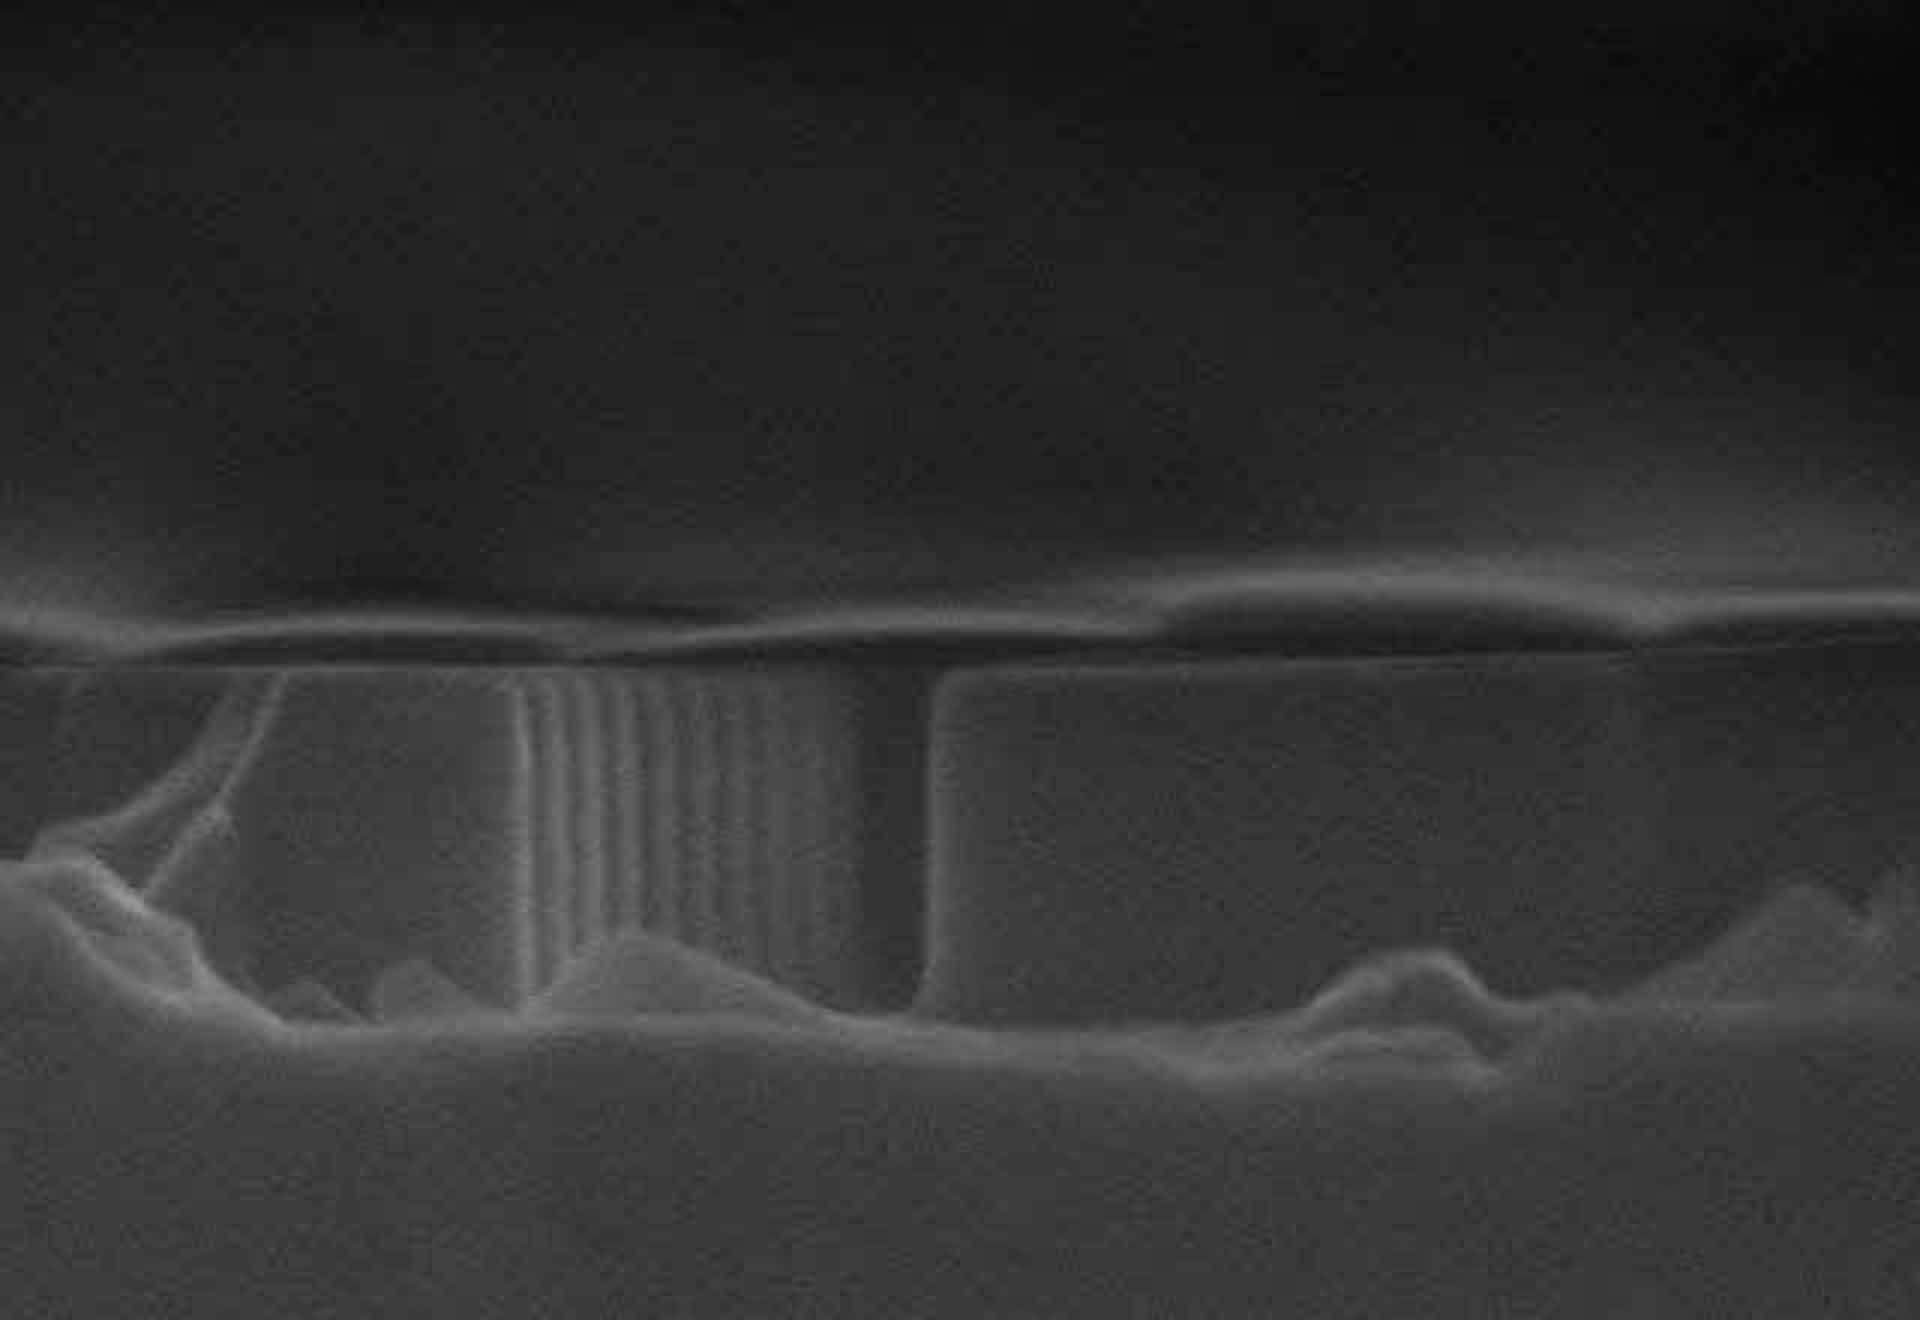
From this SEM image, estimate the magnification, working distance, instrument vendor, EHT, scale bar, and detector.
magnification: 80 K X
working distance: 15.3 mm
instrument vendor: Hitachi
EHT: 15 kV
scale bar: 500 nm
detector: SE(M)